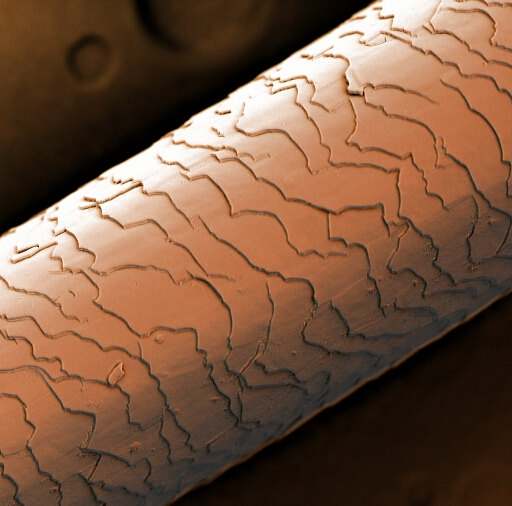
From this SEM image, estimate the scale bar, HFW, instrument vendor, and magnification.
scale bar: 50000 nm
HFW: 101 µm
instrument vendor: Phenom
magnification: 2.65 K X